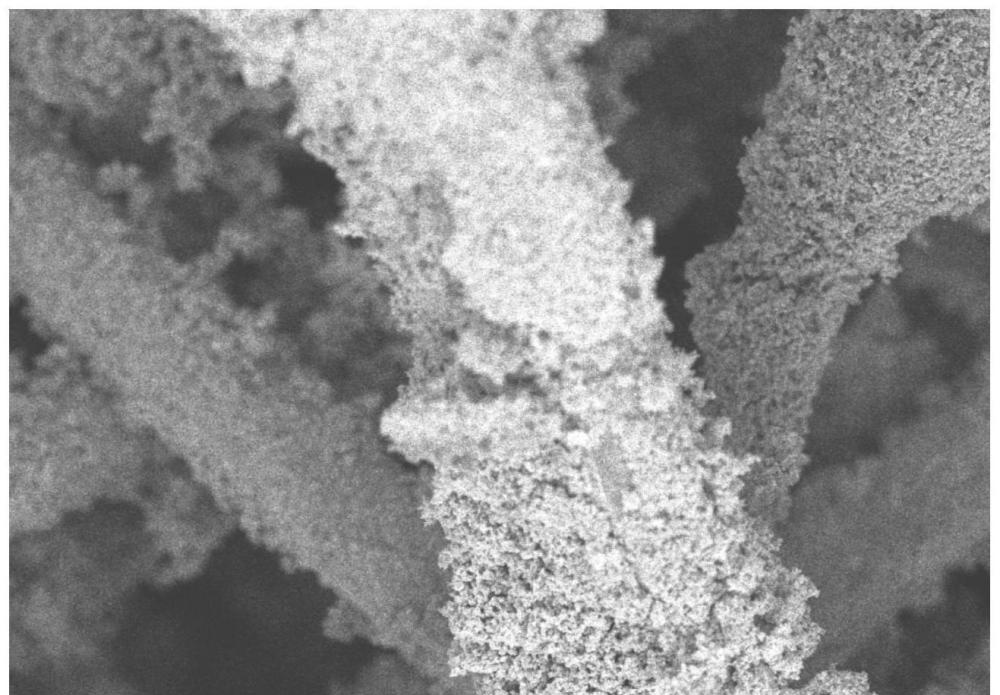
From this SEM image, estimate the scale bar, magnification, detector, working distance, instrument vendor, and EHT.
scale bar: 50000 nm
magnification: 1 K X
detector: UD(+LD)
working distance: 7.8 mm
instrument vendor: Hitachi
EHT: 5 kV